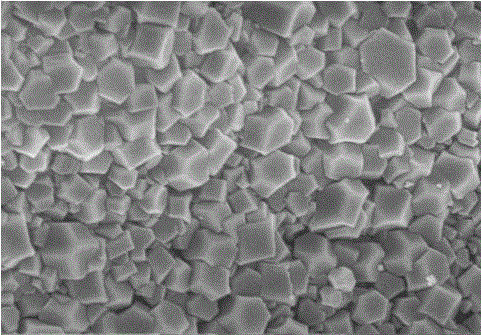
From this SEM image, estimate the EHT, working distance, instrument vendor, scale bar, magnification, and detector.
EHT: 5 kV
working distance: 11.2 mm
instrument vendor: Hitachi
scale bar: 2000 nm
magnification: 20 K X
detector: SE(M)